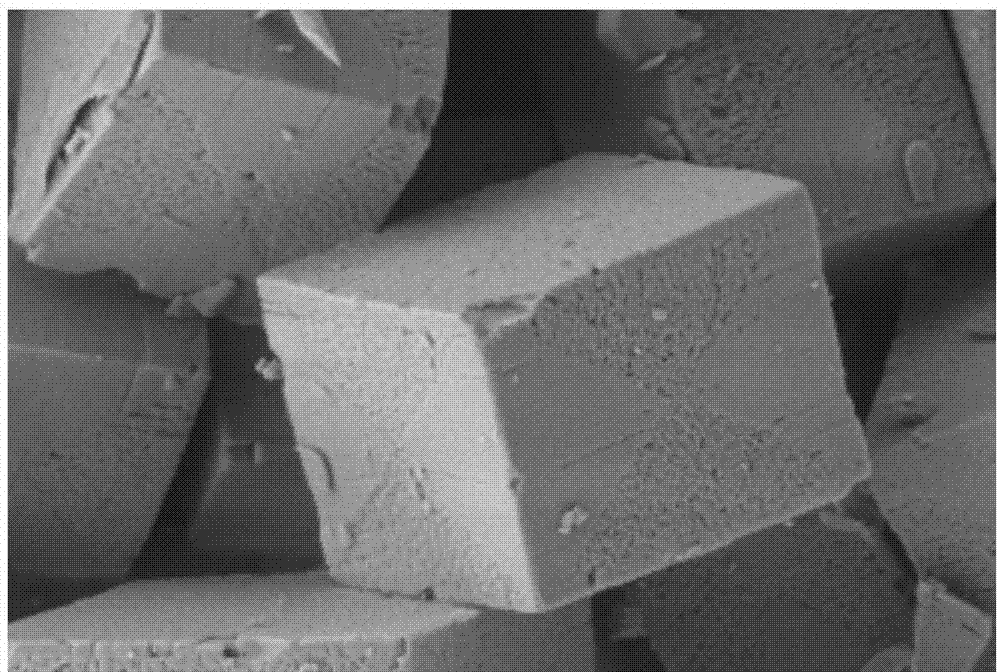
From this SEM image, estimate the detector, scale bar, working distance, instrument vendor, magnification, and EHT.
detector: SE2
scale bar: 1000 nm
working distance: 6.6 mm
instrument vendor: Zeiss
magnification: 10 K X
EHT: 2 kV